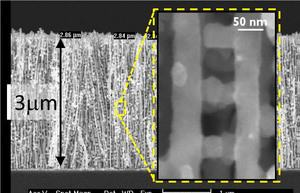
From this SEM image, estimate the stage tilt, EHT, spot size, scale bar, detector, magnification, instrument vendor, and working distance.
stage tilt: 0°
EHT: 5 kV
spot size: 3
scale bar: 1000 nm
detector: TLD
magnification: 39.282 K X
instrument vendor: FEI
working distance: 4.1 mm